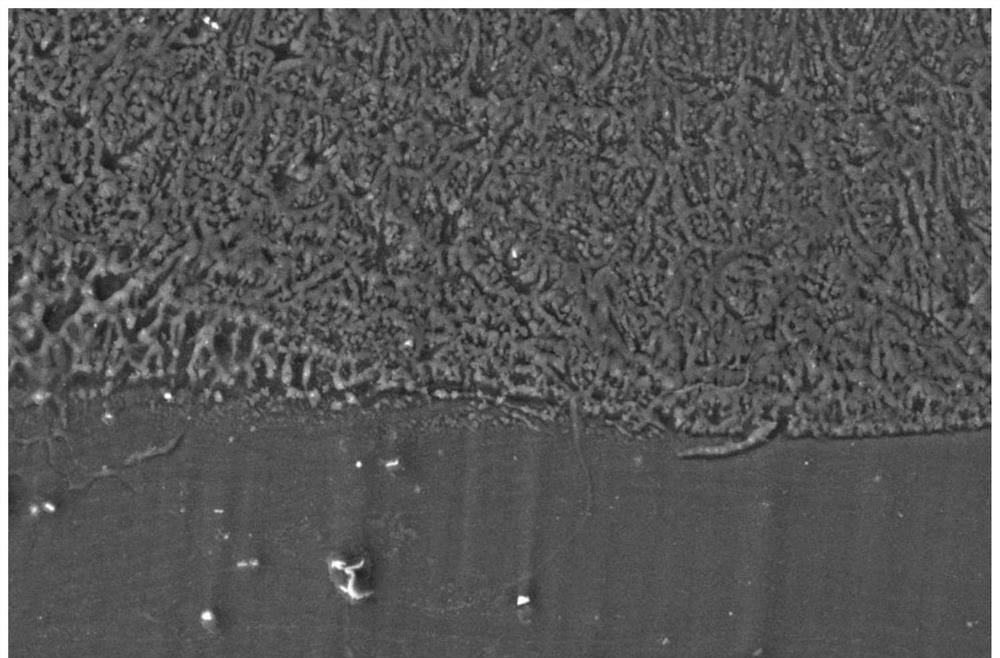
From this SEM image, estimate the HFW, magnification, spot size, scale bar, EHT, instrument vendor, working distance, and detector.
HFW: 207 µm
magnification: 2 K X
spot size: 5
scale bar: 50000 nm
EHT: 20 kV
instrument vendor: FEI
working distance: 11.5 mm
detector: ETD